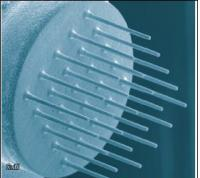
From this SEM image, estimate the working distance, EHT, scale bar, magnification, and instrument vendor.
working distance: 17.9 mm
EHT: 25 kV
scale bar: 200000 nm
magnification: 0.12 K X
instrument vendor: other